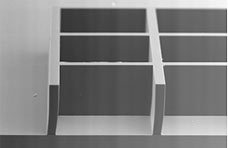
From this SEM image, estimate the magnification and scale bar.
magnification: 0.1 K X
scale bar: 200000 nm